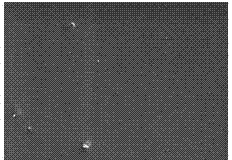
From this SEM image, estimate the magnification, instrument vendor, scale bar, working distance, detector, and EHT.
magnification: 0.55 K X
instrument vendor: Hitachi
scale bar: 100000 nm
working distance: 10.4 mm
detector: SE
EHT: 15 kV